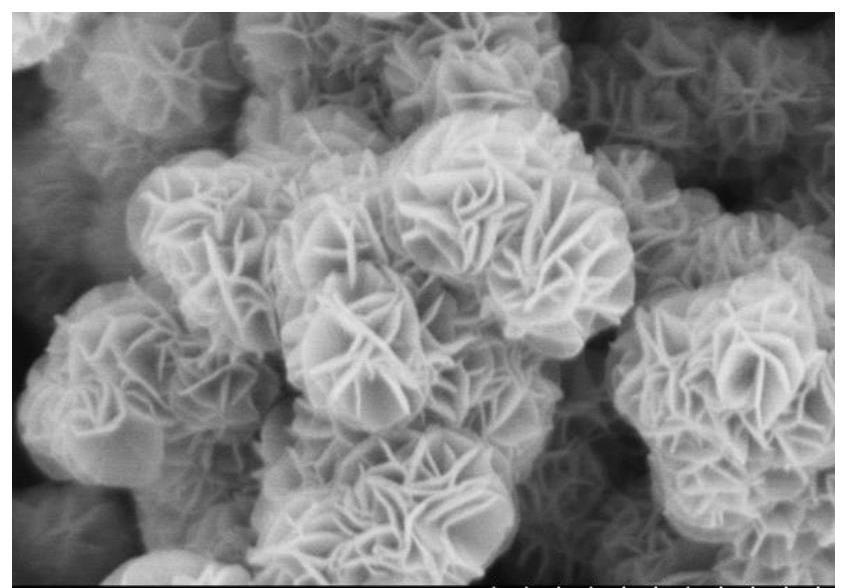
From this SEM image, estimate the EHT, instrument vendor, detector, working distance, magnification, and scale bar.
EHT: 5 kV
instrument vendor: Hitachi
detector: SE(M)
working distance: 8.5 mm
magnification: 50 K X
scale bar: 1000 nm